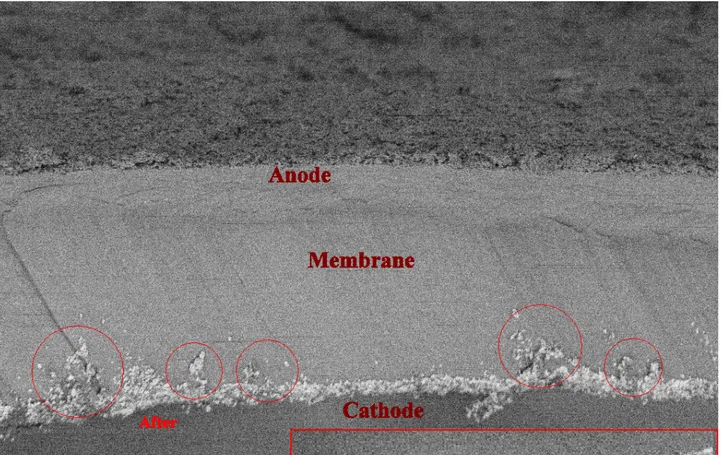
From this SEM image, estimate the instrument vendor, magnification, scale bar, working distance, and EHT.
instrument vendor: Zeiss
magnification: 5 K X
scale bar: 10000 nm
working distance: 8.3 mm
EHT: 1.7 kV